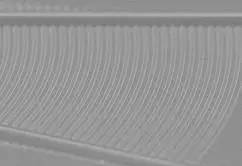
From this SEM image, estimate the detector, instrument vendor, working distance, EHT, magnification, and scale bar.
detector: SE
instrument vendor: Hitachi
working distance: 0.8 mm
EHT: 3 kV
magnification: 10 K X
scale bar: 5000 nm